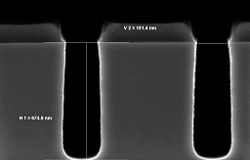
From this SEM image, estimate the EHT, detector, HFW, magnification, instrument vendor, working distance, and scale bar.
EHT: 2 kV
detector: InLens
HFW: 1.44 µm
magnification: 208.49 K X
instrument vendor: Zeiss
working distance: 3.4 mm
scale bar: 100 nm